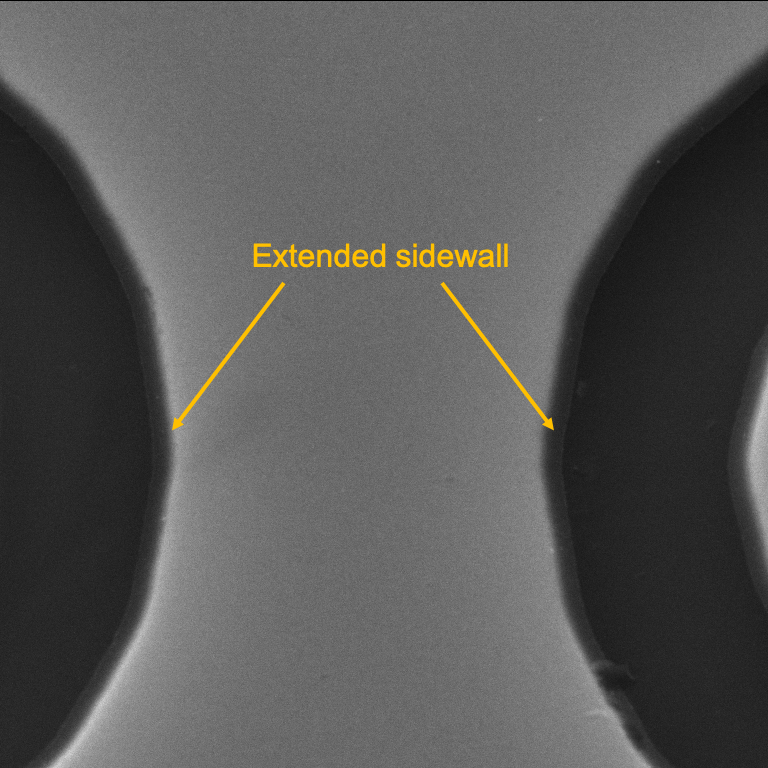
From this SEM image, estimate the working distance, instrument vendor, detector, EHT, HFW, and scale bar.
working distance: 10.04 mm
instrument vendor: Tescan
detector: SE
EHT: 28 kV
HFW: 20.6 µm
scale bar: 5000 nm